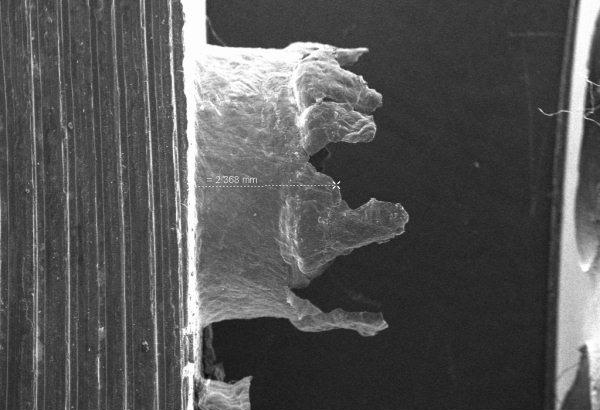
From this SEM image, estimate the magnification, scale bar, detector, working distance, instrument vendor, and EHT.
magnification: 0.032 K X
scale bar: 1000000 nm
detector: SE1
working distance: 25 mm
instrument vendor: Zeiss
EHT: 20 kV